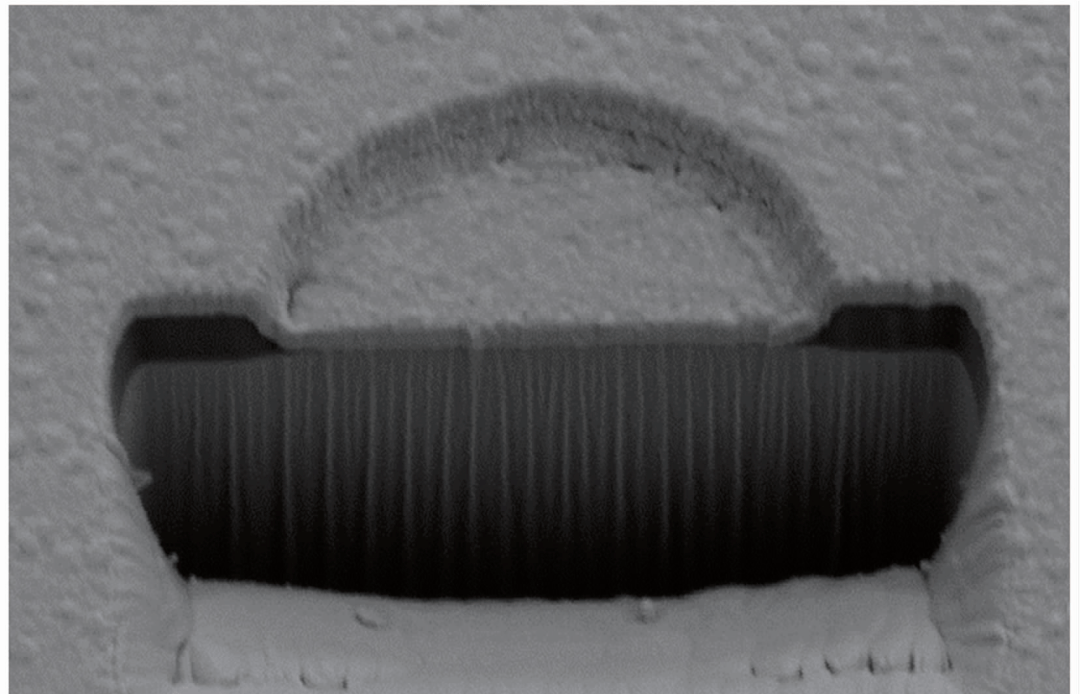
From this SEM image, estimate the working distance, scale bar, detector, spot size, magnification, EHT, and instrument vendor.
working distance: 5.2 mm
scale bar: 2000 nm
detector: SE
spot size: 3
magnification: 10 K X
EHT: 5 kV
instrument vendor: FEI